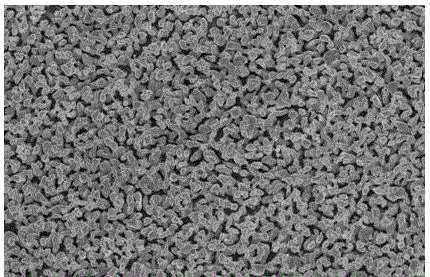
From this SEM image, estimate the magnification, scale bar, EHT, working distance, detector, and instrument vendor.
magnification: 20 K X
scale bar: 10000 nm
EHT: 15 kV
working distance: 5 mm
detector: SE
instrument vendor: FEI